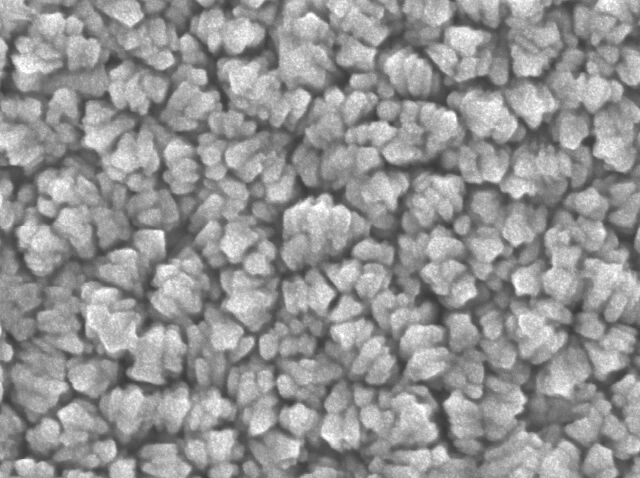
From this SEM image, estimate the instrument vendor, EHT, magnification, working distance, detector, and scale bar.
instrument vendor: JEOL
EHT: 5 kV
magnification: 200 K X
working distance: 2 mm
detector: SEI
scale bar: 100 nm